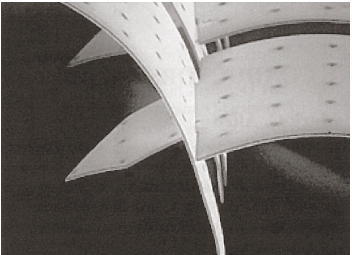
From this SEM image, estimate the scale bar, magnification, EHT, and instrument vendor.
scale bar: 100000 nm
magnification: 0.366 K X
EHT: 20 kV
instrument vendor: other